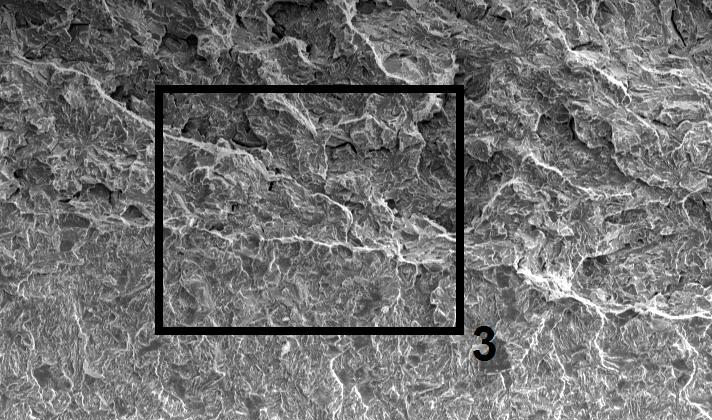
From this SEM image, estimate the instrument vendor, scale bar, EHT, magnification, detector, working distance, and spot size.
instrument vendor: FEI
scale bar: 200000 nm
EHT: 20 kV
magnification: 0.1 K X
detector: SE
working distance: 29.3 mm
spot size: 4.4